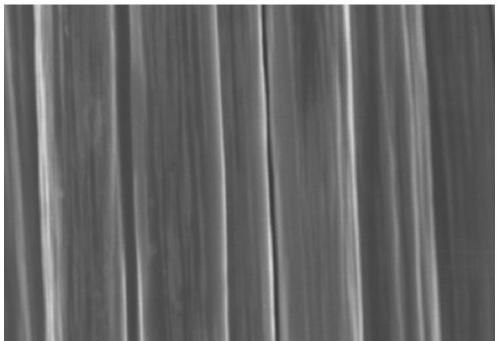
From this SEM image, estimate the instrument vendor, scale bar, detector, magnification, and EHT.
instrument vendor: Hitachi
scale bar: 10000 nm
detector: SE(M)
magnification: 3 K X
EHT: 8 kV